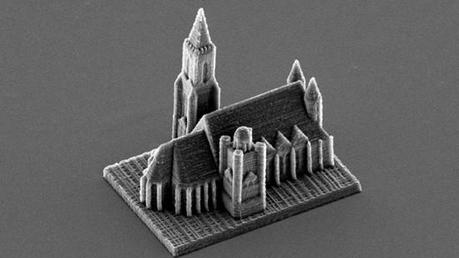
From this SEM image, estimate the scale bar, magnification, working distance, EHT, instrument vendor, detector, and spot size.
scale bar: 50000 nm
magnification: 0.628 K X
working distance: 20.1 mm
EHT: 12 kV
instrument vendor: FEI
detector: SE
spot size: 4